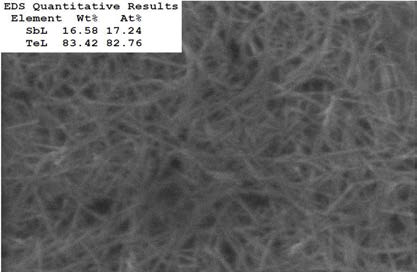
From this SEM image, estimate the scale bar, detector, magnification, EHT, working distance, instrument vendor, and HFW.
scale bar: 500 nm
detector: InBeam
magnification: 100 K X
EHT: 20 kV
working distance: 14.42 mm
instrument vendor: Tescan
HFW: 2.17 µm